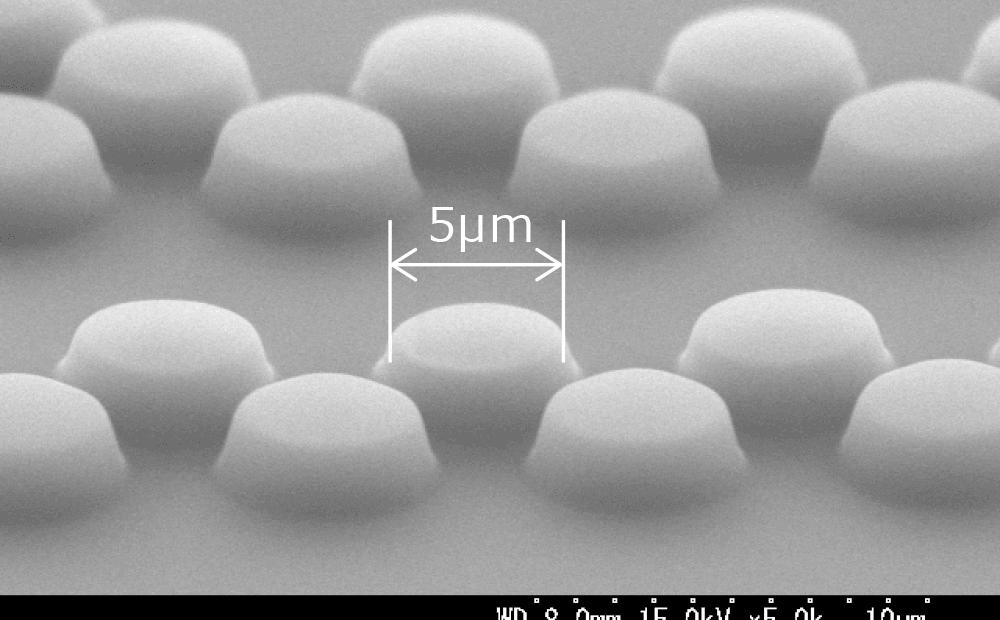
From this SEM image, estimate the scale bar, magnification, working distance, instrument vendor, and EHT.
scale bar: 10000 nm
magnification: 5 K X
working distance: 8 mm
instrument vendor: Hitachi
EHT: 15 kV